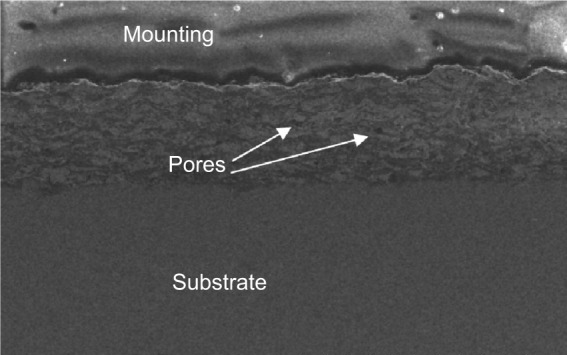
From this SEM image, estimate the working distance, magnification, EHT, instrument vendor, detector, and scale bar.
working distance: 10.5 mm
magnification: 0.1 K X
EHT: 15 kV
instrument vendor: Hitachi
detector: SE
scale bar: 500000 nm